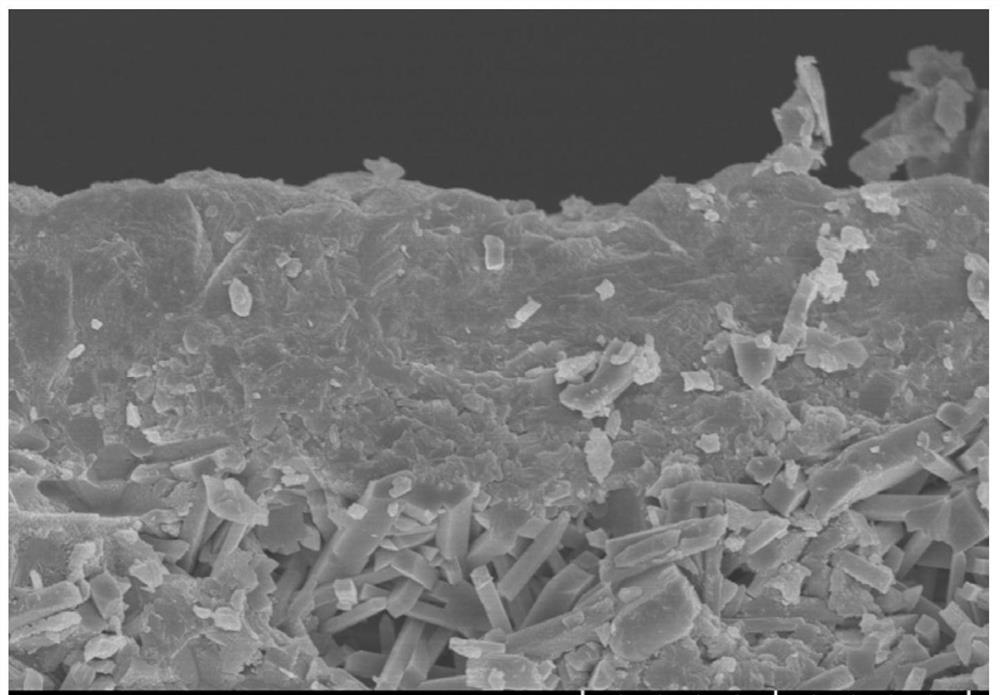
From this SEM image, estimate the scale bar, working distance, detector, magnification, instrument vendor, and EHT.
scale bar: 5000 nm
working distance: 9.9 mm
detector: SE(UL)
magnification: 10 K X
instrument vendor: Hitachi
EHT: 5 kV